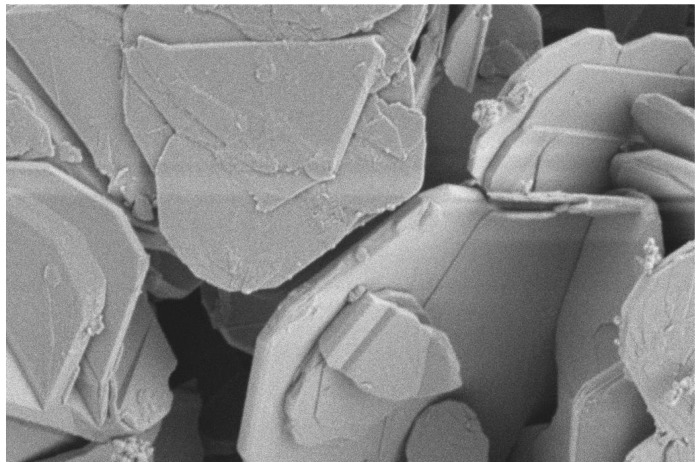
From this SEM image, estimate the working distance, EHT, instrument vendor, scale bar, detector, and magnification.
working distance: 8.6 mm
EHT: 2 kV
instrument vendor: Zeiss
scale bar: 1000 nm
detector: SE2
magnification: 10 K X